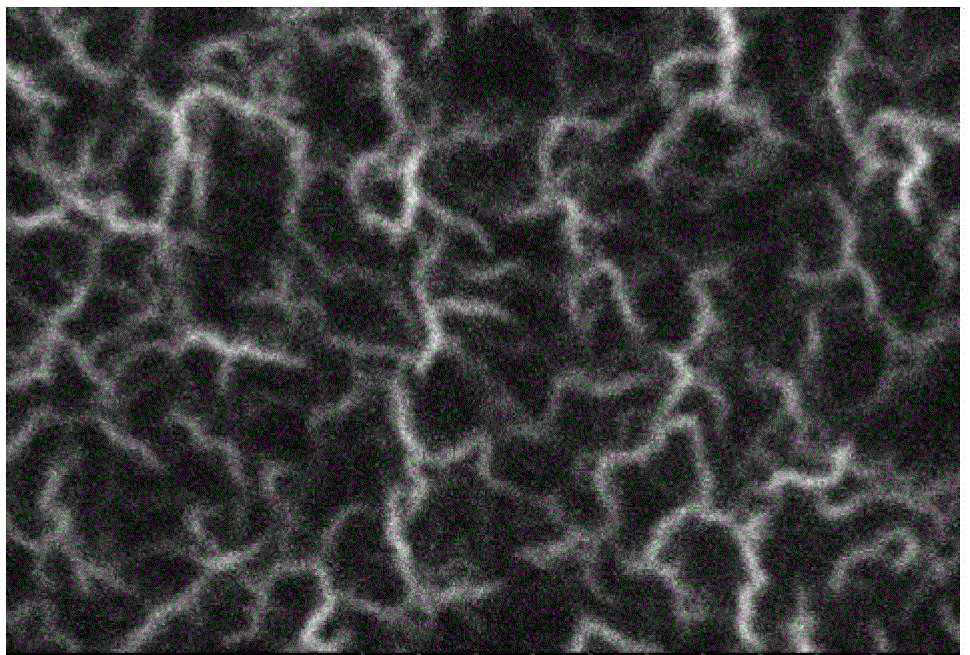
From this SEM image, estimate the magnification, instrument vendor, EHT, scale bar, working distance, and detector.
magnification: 50 K X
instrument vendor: Hitachi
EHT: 15 kV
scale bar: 1000 nm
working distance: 4.8 mm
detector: SE